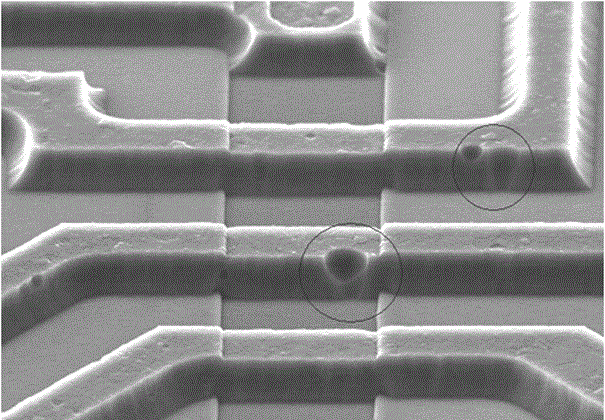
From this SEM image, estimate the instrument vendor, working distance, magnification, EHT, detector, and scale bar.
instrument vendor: Hitachi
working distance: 14.7 mm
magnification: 2 K X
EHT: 15 kV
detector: SE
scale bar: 20000 nm